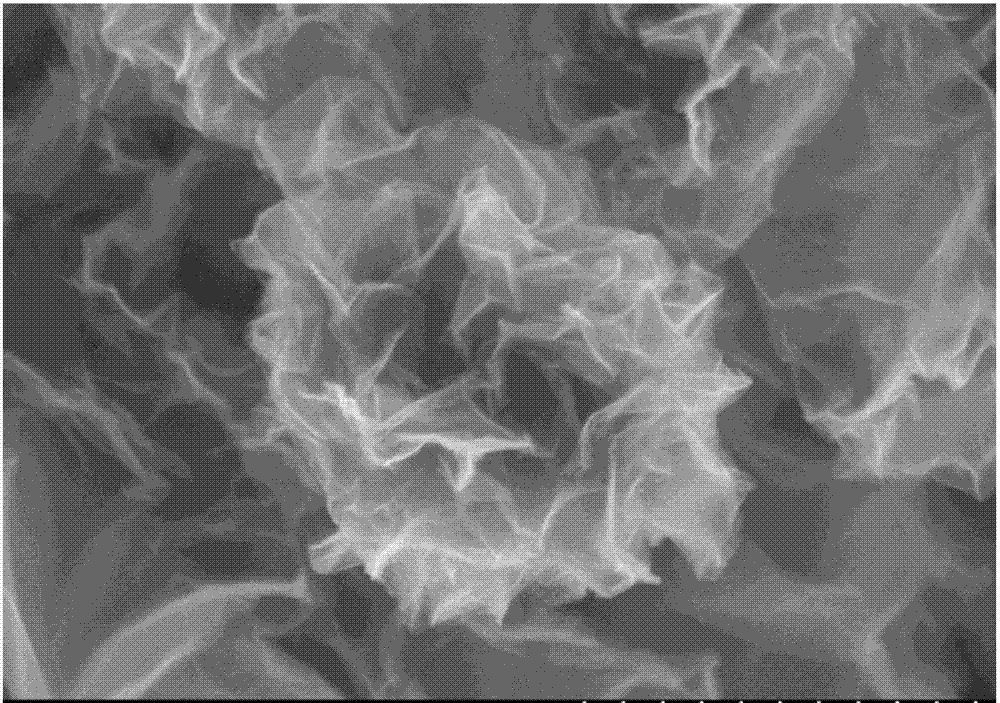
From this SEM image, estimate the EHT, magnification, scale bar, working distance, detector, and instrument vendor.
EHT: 3 kV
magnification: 50 K X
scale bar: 1000 nm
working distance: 8.9 mm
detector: SE(M)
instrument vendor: Hitachi